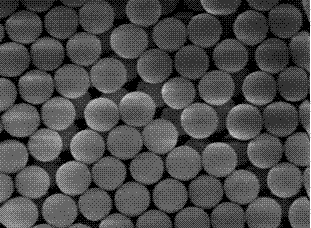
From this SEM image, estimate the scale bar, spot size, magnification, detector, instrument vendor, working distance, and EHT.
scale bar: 500 nm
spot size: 3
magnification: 40 K X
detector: TLD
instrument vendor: FEI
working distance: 4.9 mm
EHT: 5 kV